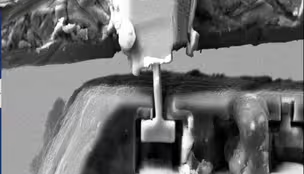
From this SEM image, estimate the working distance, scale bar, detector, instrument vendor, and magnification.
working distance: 8.4 mm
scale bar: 10000 nm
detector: SE2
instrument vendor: Zeiss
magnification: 1.46 K X